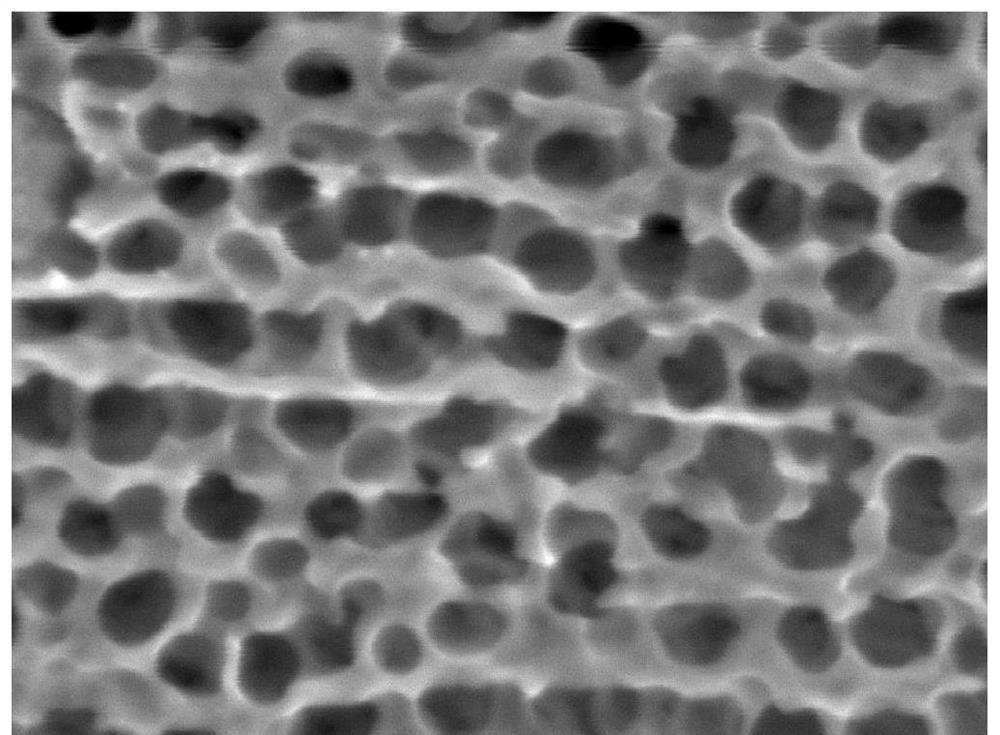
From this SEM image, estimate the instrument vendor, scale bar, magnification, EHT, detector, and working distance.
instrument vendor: JEOL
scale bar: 100 nm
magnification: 150 K X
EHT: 10 kV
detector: LED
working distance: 10 mm